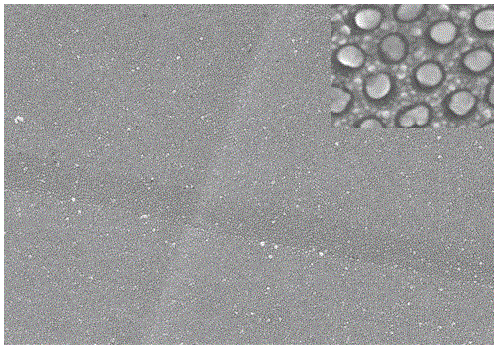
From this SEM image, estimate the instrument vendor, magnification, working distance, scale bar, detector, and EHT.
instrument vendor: JEOL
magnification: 10 K X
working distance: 12.8 mm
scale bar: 1000 nm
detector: SEI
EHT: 15 kV